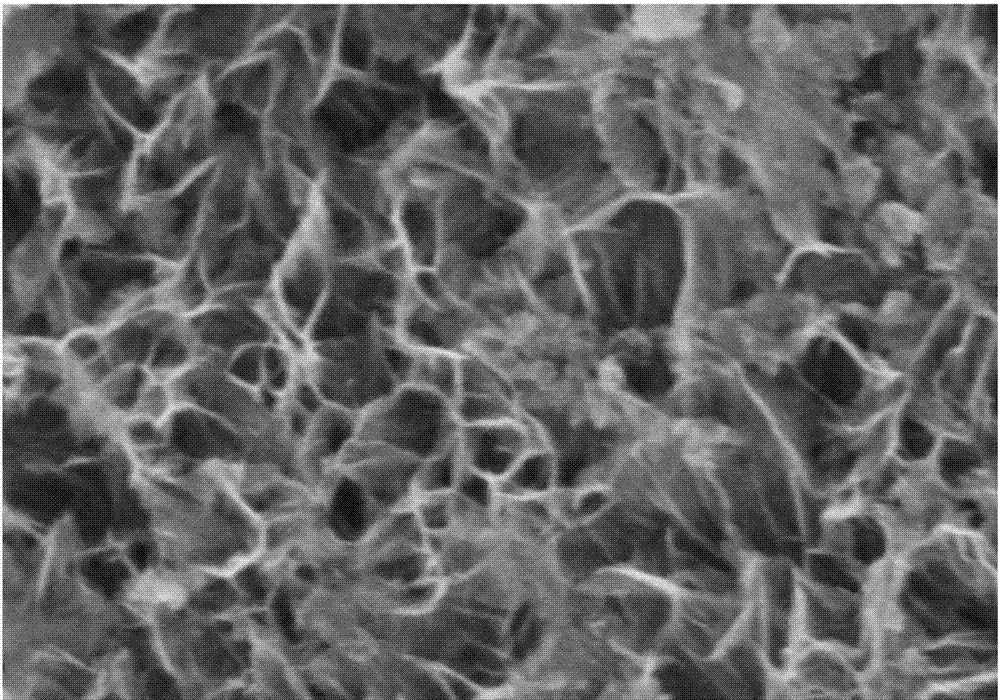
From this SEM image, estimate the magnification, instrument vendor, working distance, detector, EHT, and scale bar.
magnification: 80 K X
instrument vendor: Hitachi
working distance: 7.9 mm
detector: SE(M)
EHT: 5 kV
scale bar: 500 nm